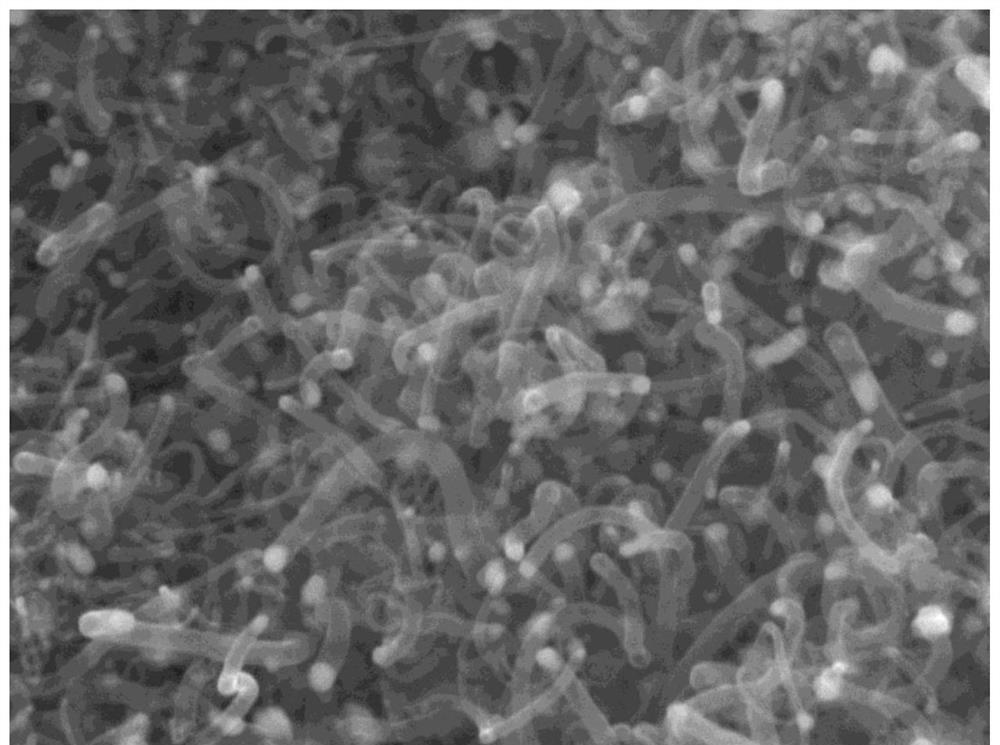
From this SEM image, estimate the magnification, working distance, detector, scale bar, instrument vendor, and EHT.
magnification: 40 K X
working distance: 10.2 mm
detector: SEI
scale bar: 100 nm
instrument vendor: JEOL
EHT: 15 kV